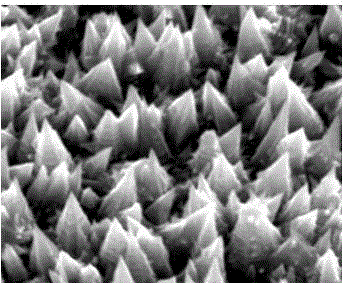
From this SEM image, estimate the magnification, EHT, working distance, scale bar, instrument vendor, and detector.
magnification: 80 K X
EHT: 20 kV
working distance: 5.9 mm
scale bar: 1000 nm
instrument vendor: FEI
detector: SE